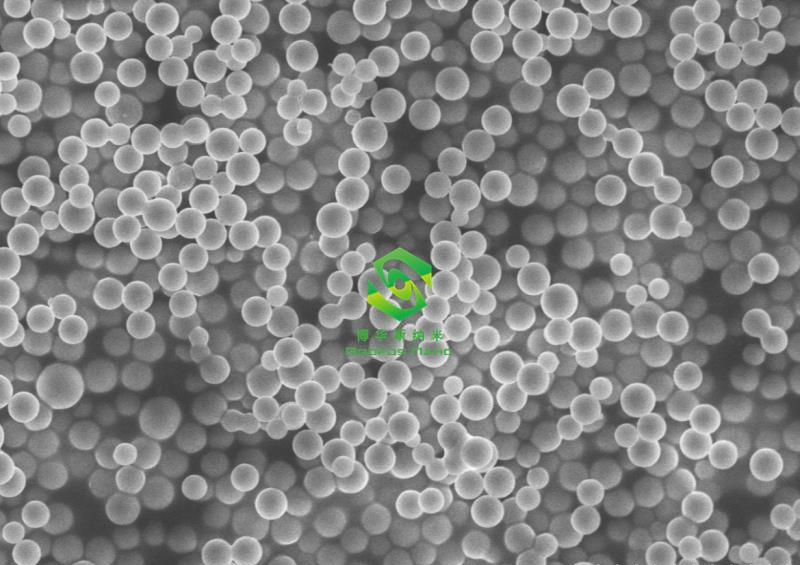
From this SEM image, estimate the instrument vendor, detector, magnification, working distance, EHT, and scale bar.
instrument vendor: Hitachi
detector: SE(M)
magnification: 6 K X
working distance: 9.5 mm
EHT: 5 kV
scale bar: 5000 nm